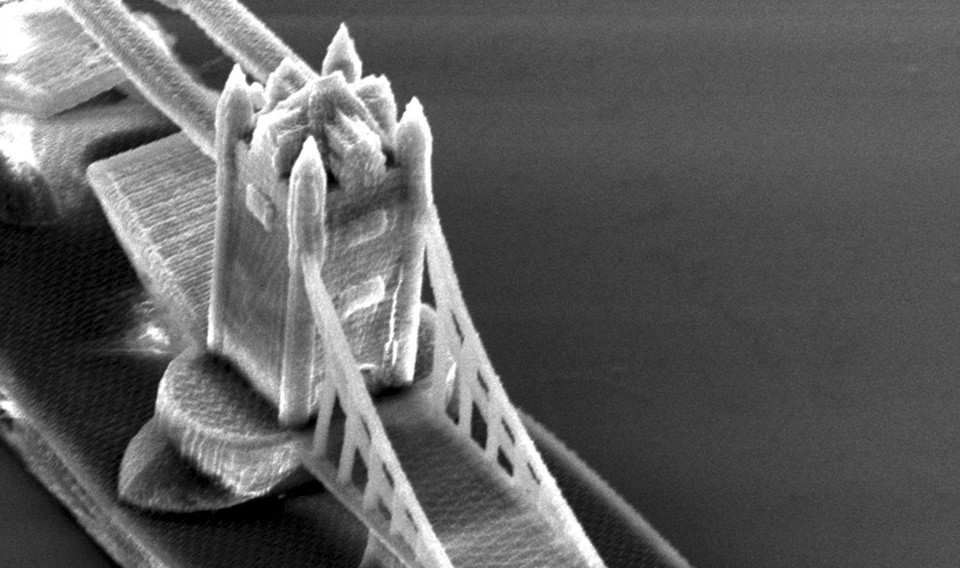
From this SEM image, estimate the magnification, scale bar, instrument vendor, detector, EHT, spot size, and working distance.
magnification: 0.969 K X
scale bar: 20000 nm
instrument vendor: FEI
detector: SE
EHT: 15 kV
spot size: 4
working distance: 27.1 mm